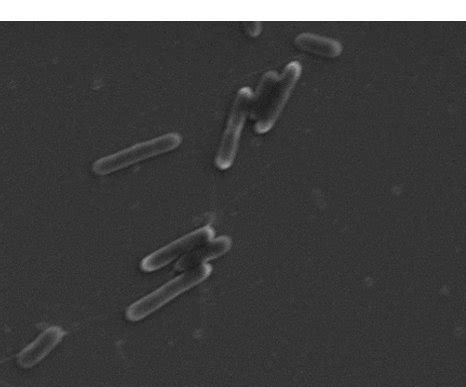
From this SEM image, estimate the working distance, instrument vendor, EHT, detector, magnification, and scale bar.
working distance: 7.4 mm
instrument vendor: Hitachi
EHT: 5 kV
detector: SE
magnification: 4.7 K X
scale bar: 10000 nm